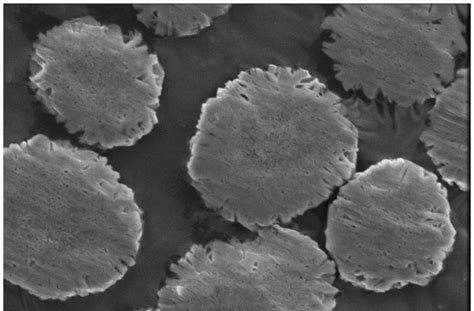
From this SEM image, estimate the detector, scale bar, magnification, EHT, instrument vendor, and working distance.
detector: ETD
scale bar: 5000 nm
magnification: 20 K X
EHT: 5 kV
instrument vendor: FEI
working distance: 9.5 mm